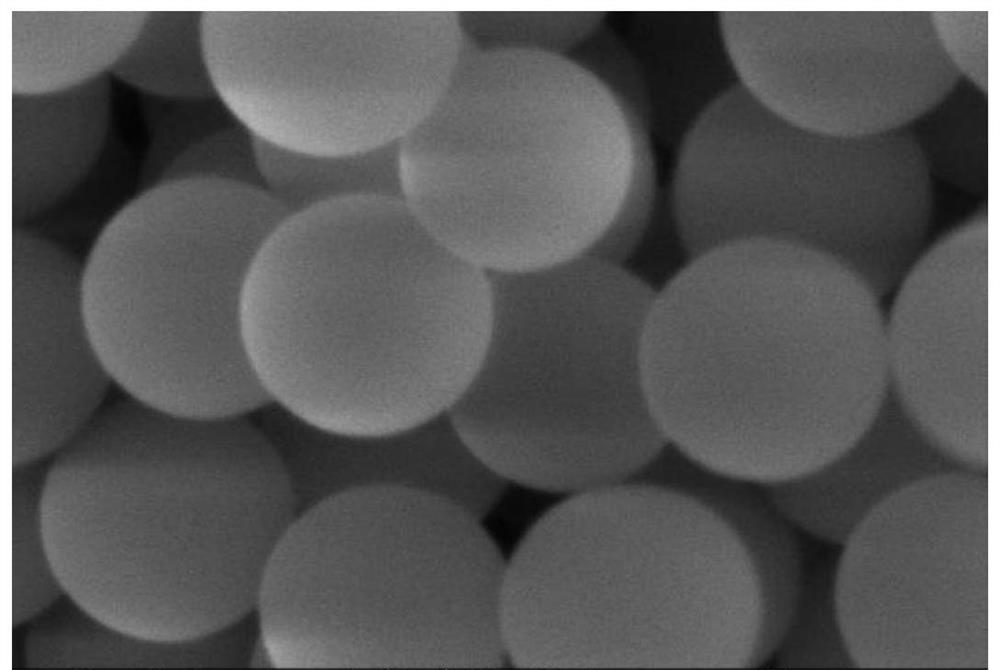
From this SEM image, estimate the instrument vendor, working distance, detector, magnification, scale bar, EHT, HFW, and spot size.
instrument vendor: FEI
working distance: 11.9 mm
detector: ETD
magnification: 240 K X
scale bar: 500 nm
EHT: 5 kV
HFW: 1.73 µm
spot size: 2.5